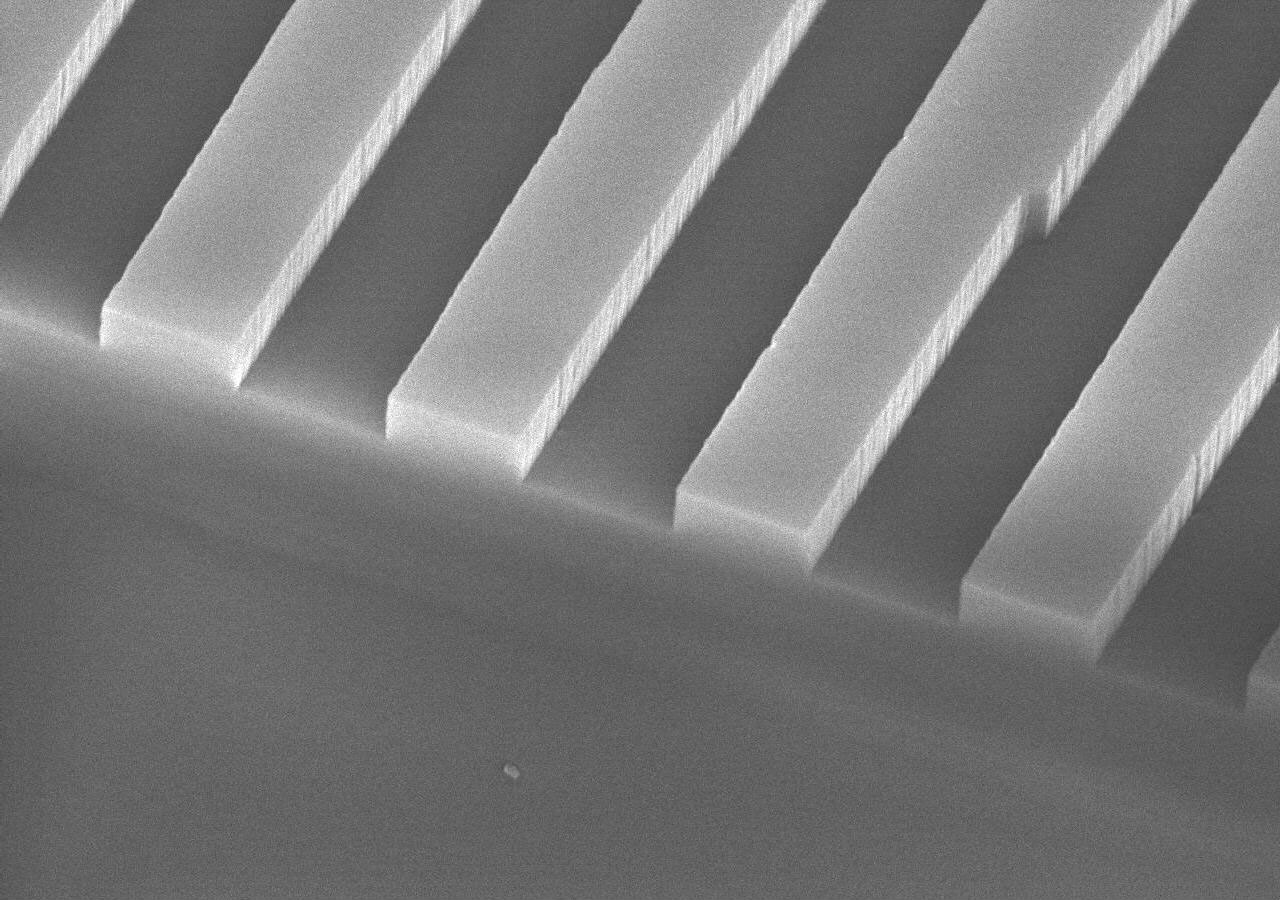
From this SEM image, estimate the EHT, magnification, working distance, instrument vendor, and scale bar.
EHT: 15 kV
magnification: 10 K X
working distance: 13.8 mm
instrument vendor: JEOL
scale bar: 1000 nm